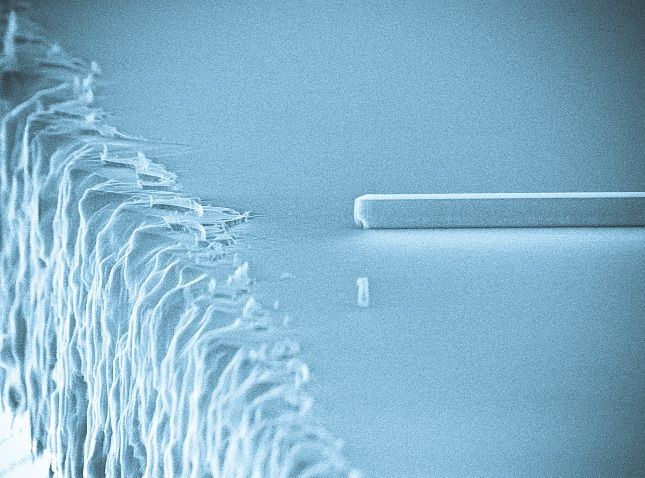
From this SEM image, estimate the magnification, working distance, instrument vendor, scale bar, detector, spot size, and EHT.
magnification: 10.011 K X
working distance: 19.4 mm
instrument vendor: FEI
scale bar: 2000 nm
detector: SE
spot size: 3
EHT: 30 kV